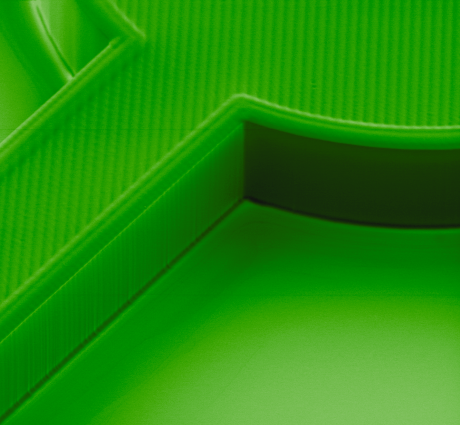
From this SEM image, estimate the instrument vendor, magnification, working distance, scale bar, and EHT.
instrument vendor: FEI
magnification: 0.259 K X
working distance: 12.8 mm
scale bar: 500000 nm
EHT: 15 kV